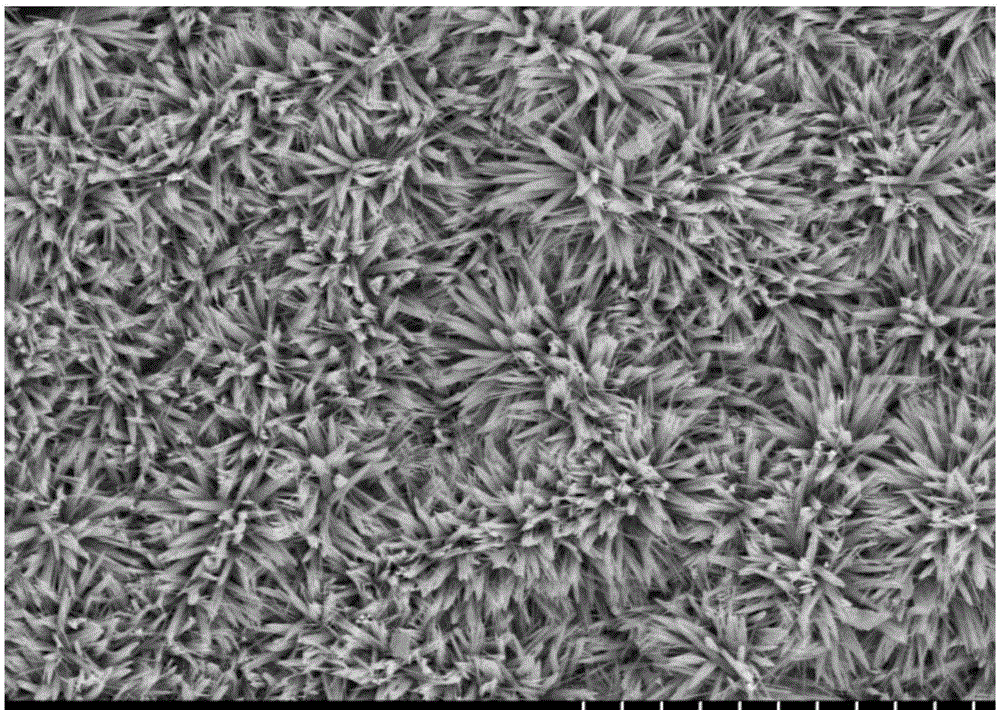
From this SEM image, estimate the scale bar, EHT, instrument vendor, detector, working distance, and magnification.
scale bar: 50000 nm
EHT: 15 kV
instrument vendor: Hitachi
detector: SE(M)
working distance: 11.7 mm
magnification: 1 K X